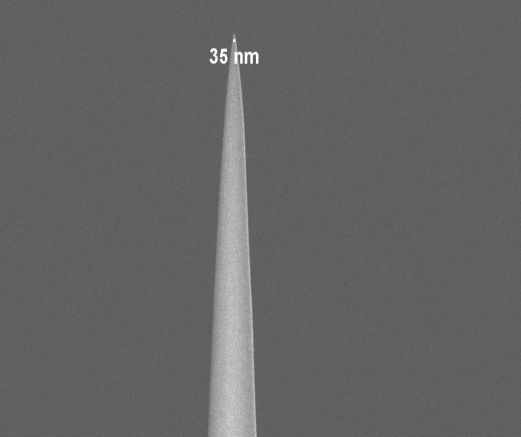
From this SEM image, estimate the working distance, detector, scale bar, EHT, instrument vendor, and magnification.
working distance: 4.9 mm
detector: InLens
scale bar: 2000 nm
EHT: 2 kV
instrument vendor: Zeiss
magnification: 20 K X